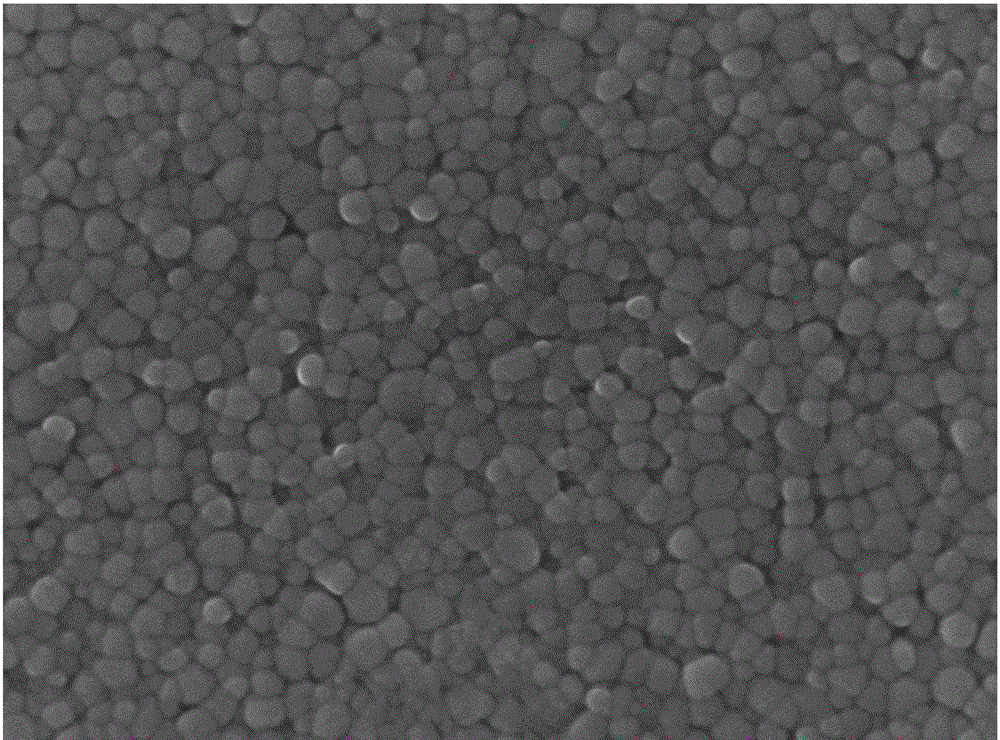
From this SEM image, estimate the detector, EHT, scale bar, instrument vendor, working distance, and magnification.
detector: SEI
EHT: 15 kV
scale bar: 100 nm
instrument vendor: JEOL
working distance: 8.3 mm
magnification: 50 K X